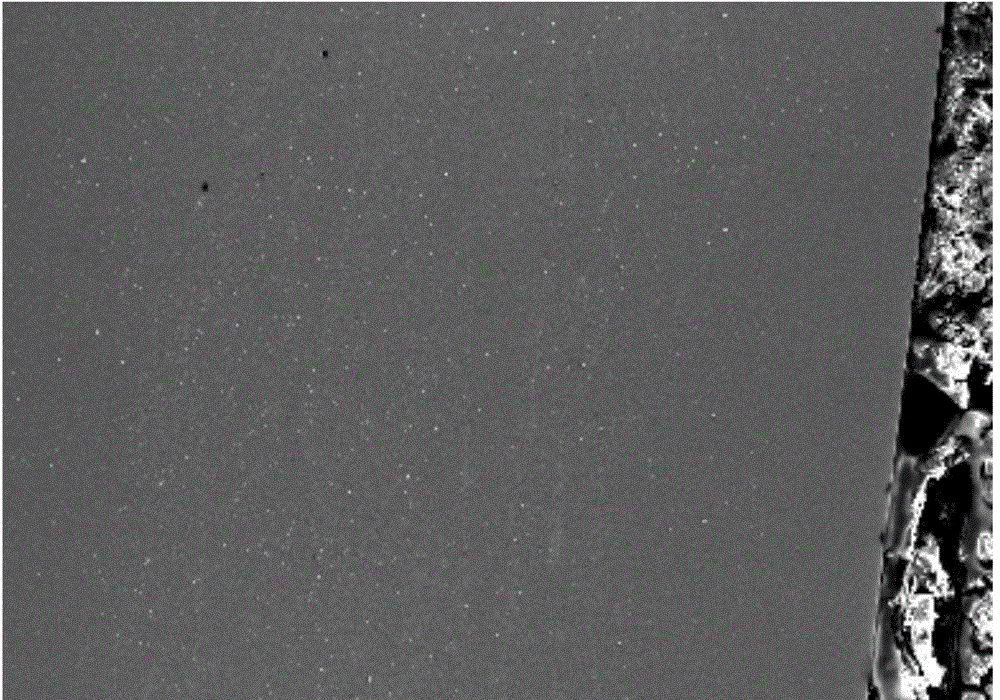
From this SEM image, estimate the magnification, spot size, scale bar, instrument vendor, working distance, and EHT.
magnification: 0.05 K X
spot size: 59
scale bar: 500000 nm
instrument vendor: JEOL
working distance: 15 mm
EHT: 20 kV